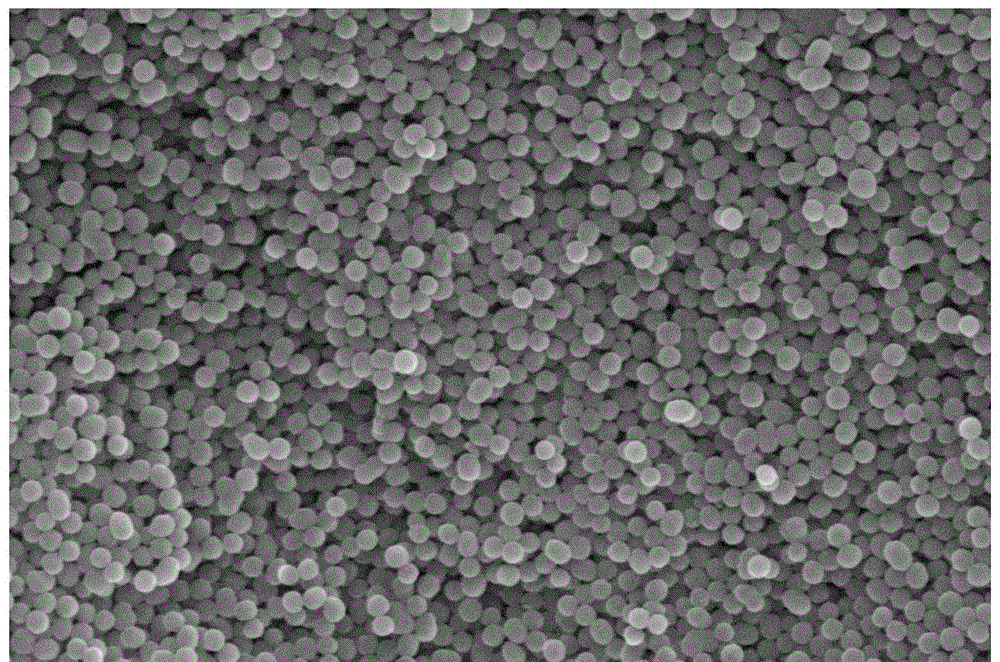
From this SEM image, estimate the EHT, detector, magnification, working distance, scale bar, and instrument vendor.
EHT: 15 kV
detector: SE2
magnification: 20 K X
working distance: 6 mm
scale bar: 200 nm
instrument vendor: Zeiss